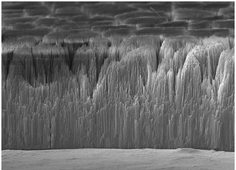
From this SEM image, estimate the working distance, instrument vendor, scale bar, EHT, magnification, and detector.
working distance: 7.8 mm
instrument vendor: JEOL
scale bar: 10000 nm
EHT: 15 kV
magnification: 1.4 K X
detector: SEI